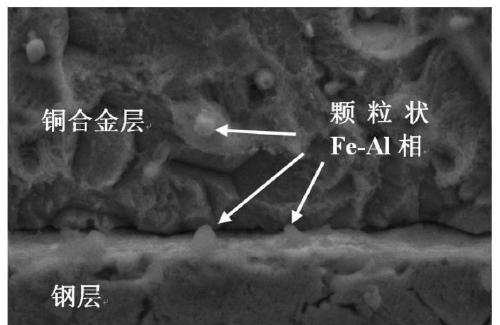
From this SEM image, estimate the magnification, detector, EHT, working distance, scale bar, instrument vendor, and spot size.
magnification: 10 K X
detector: SE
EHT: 20 kV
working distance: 6.7 mm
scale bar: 5000 nm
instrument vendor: FEI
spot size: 4